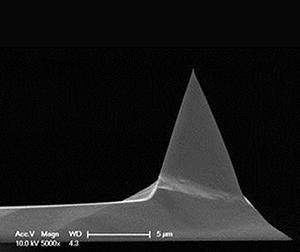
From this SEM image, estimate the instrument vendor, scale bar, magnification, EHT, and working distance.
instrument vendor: FEI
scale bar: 5000 nm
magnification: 5 K X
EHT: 10 kV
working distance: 4.3 mm